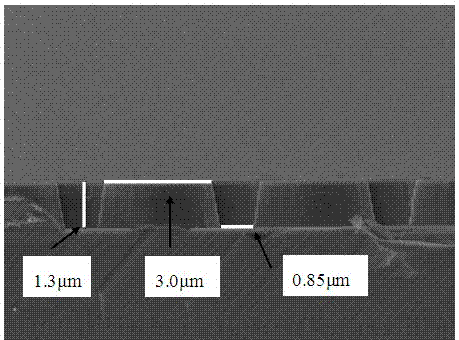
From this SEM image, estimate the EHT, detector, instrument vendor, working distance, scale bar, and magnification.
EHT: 5 kV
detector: SEI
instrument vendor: JEOL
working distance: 4.4 mm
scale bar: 1000 nm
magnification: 10 K X